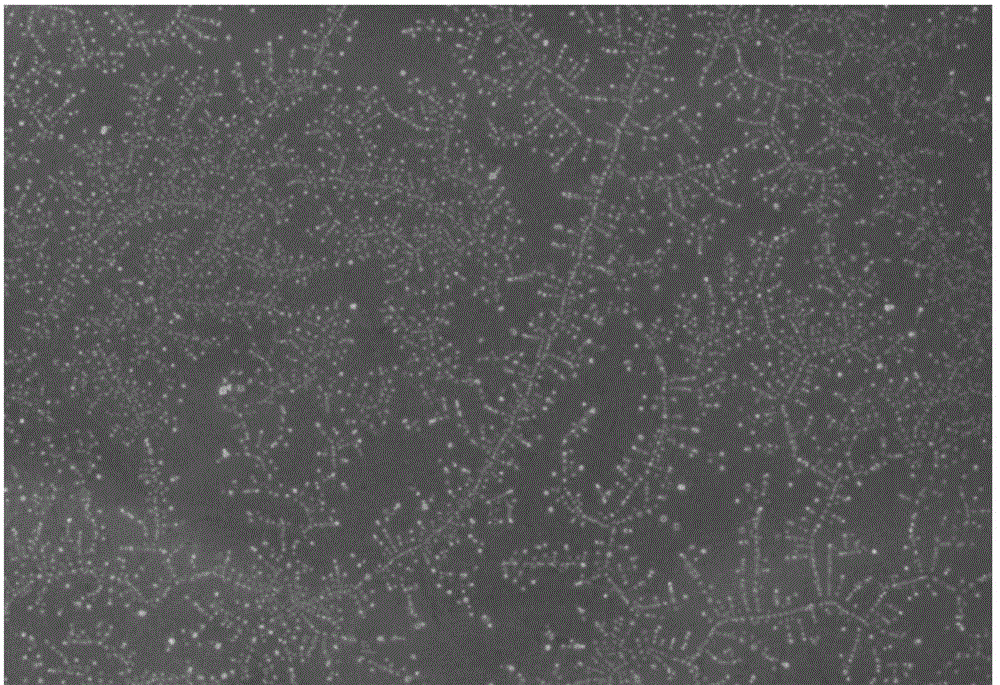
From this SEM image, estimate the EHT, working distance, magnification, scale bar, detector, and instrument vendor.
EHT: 10 kV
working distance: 10.4 mm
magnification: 0.495 K X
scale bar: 20000 nm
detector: InLens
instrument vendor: Zeiss